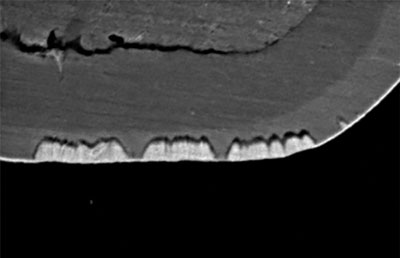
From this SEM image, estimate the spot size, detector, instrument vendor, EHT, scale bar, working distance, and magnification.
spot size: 50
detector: SEI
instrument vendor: JEOL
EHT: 15 kV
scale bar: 5000 nm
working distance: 6 mm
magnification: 5 K X